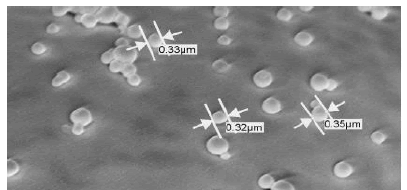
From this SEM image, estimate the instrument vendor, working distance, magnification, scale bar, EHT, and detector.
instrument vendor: JEOL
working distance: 8.4 mm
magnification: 10 K X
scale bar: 1000 nm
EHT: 5 kV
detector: SEI